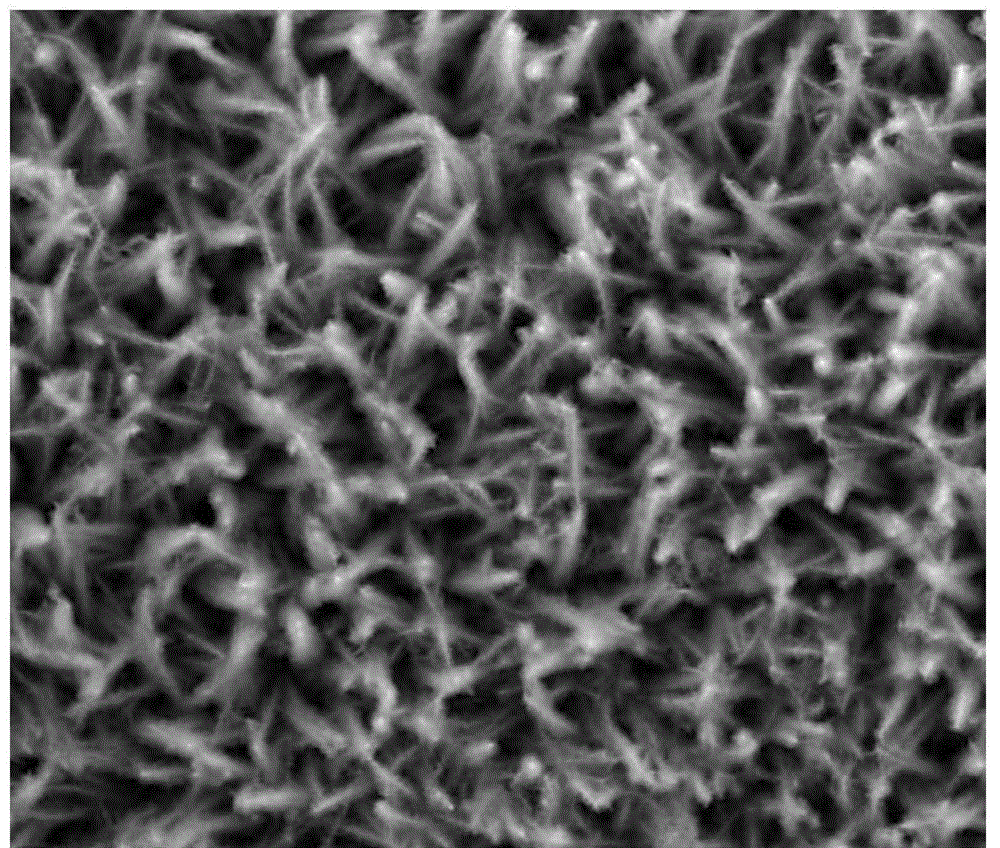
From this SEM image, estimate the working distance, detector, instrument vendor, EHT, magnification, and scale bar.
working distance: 4.2 mm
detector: TLD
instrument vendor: FEI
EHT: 10 kV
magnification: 10 K X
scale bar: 5000 nm